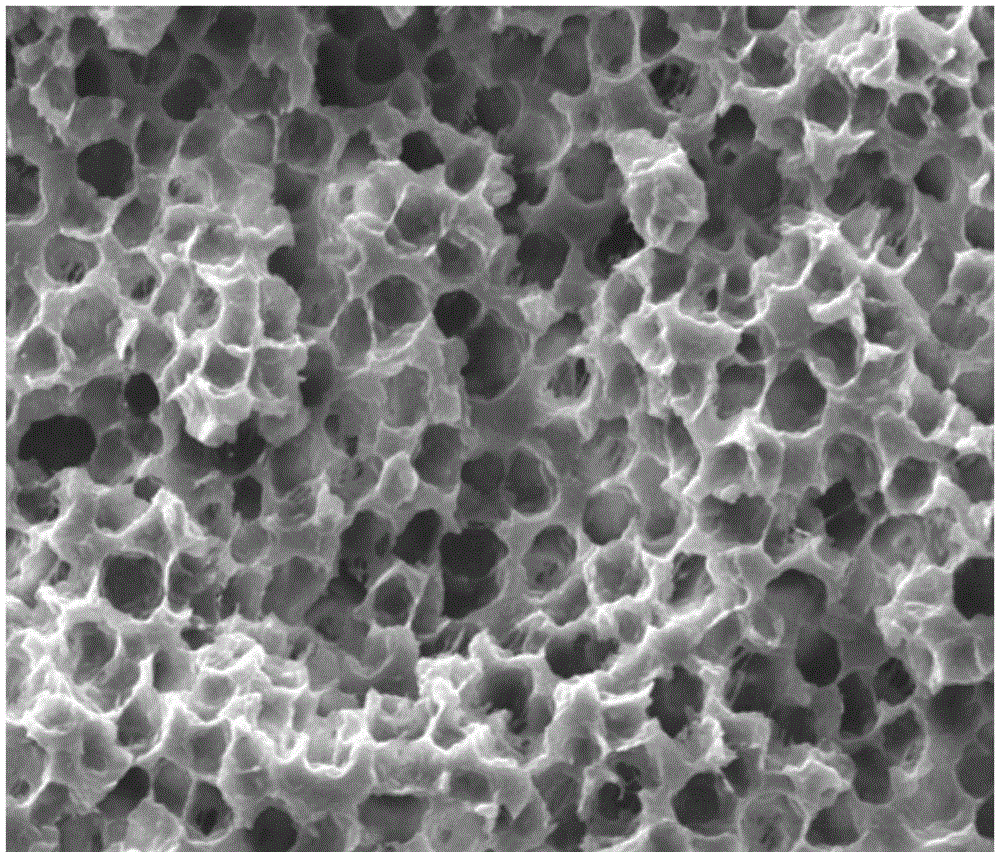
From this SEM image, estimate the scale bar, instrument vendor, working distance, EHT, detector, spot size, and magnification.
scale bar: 30000 nm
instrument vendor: FEI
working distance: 13.4 mm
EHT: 15 kV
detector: ETD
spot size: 4.5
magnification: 3 K X